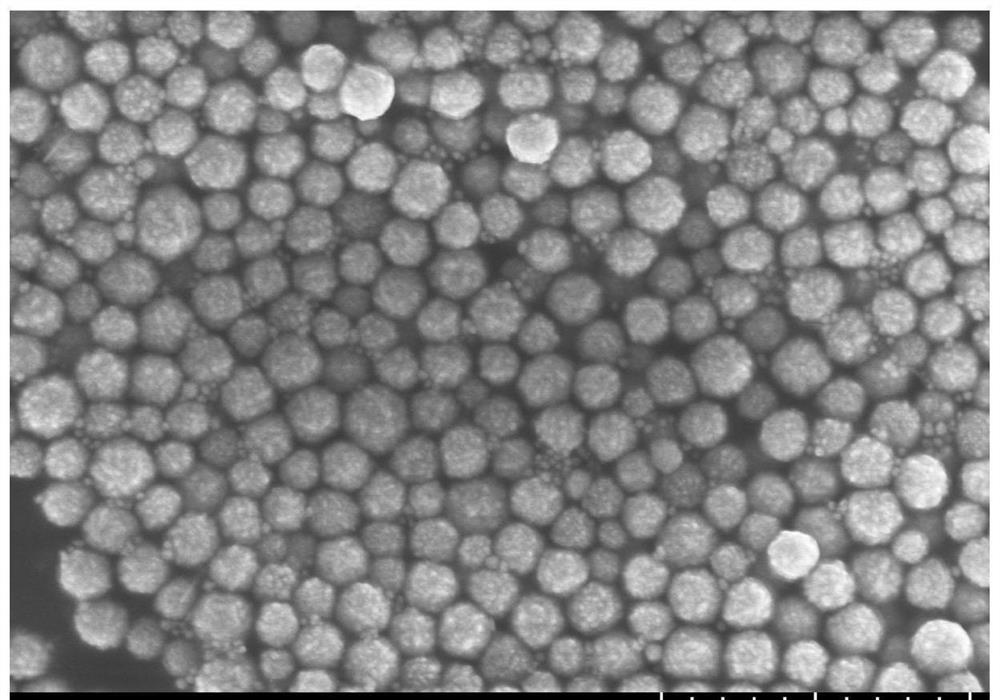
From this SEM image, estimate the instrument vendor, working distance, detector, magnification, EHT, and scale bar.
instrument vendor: Hitachi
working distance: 7.7 mm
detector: SE(TUL)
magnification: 20 K X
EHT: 5 kV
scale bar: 2000 nm